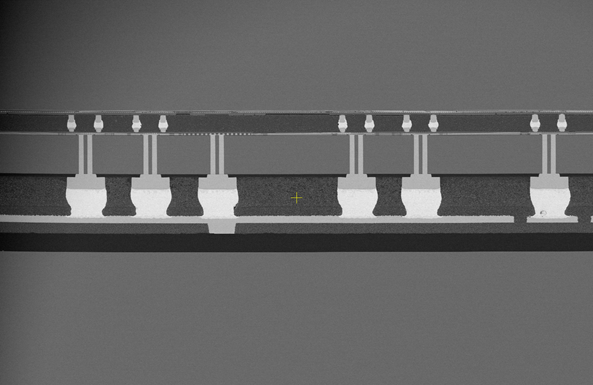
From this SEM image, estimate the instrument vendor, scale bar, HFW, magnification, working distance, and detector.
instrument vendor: Thermo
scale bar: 500000 nm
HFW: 1380 µm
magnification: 0.3 K X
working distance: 3.8 mm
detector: T1